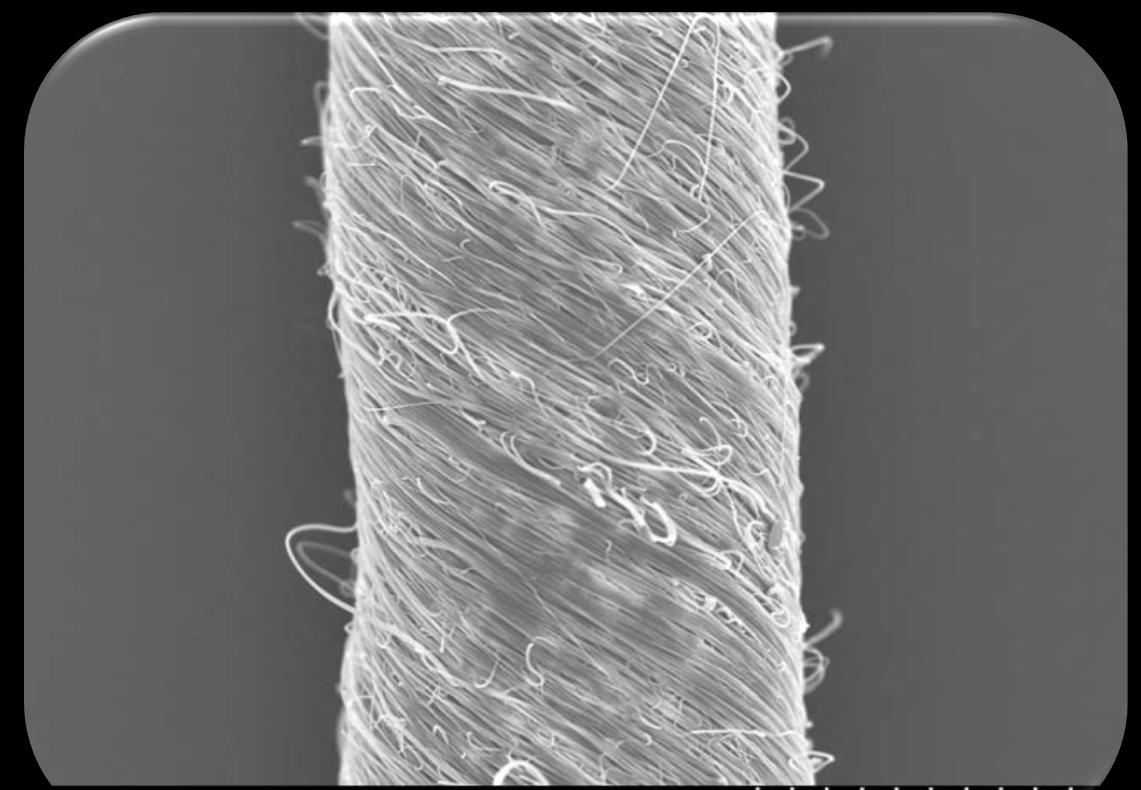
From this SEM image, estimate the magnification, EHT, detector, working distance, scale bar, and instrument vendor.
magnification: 0.8 K X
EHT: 10 kV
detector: SE(M)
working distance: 7.8 mm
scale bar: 50000 nm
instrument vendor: Hitachi